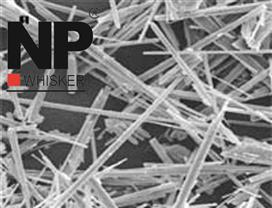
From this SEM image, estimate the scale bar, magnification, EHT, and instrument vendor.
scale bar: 30000 nm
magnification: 1 K X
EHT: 15 kV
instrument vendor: JEOL